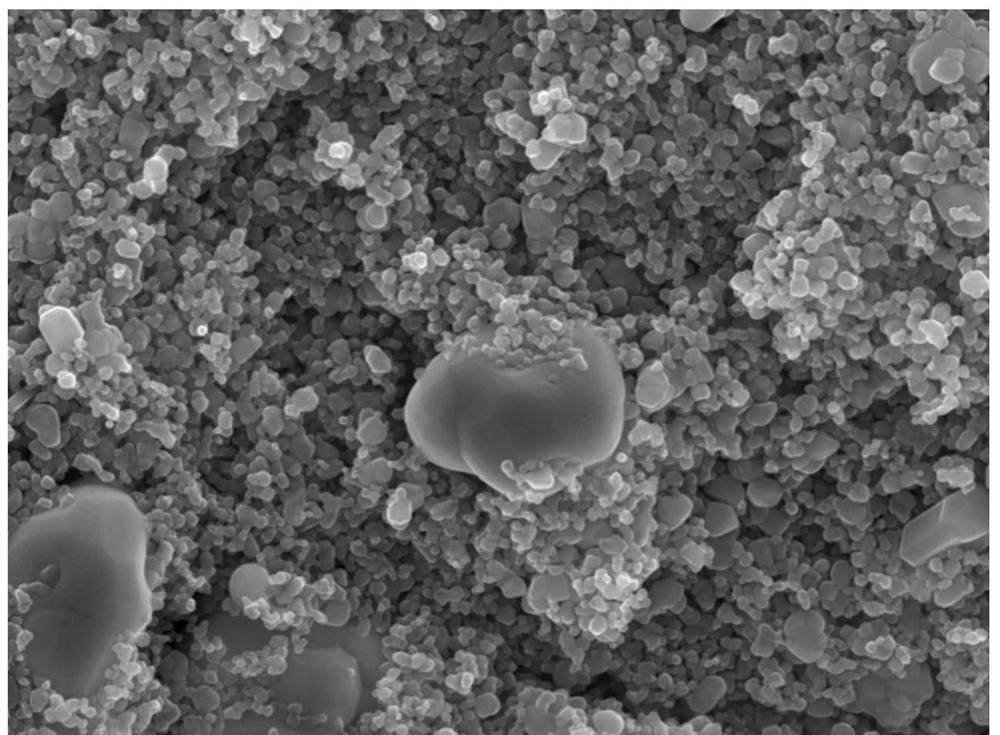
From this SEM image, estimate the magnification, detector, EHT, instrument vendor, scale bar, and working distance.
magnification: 20 K X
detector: LED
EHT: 20 kV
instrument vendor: JEOL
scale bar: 1000 nm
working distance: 10 mm